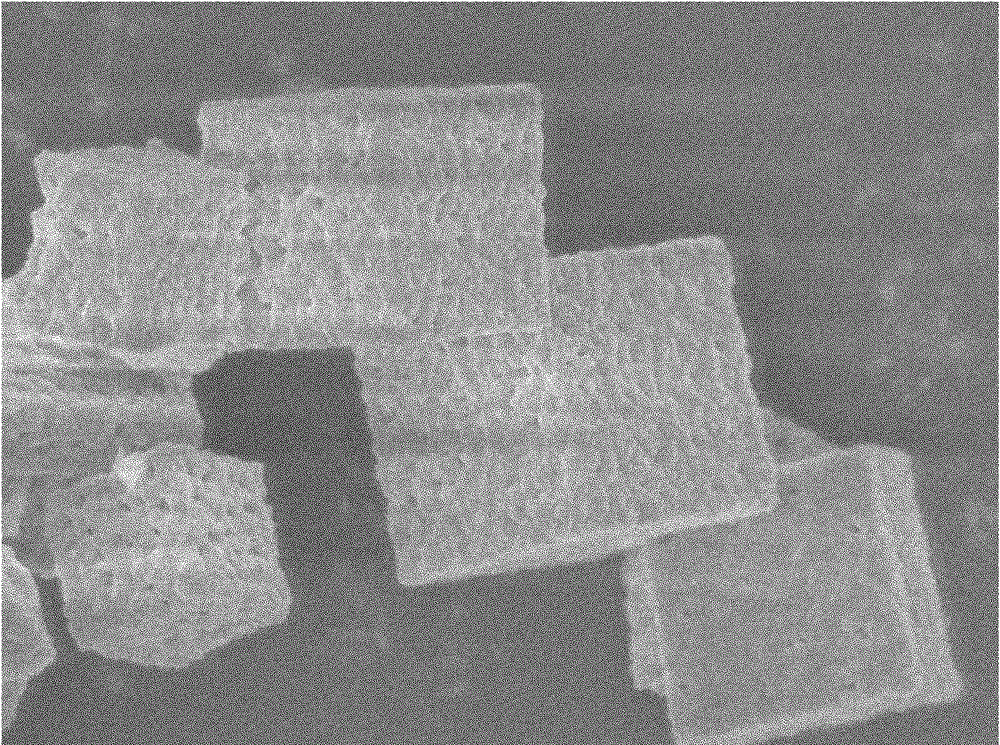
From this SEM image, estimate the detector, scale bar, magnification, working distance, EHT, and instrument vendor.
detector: SEI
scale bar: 1000 nm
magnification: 9 K X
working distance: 9.3 mm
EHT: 5 kV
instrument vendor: JEOL